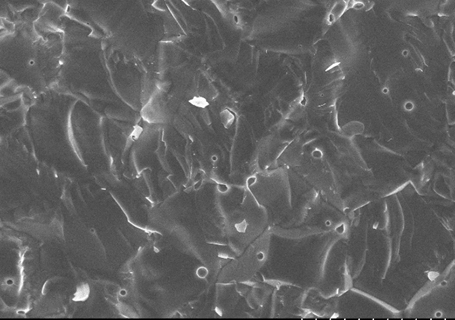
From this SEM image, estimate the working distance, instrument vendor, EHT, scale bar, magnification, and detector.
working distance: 12.3 mm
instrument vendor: Hitachi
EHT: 15 kV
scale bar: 20000 nm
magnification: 2 K X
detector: SE(U)